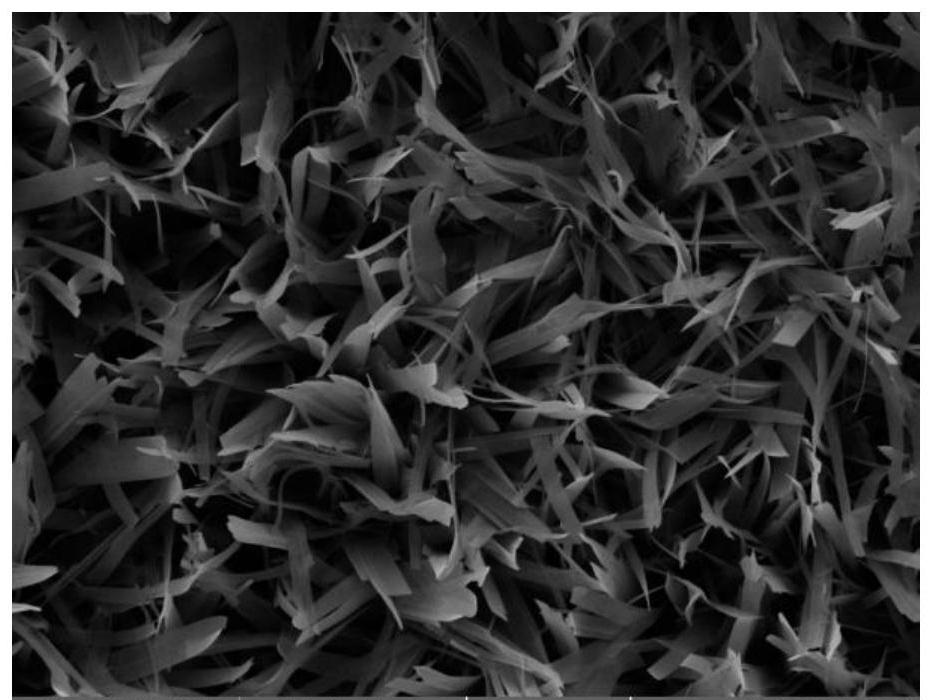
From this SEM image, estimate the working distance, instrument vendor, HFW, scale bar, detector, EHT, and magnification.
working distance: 14.58 mm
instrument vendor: Tescan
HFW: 55.4 µm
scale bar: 10000 nm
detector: SE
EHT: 20 kV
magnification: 5 K X